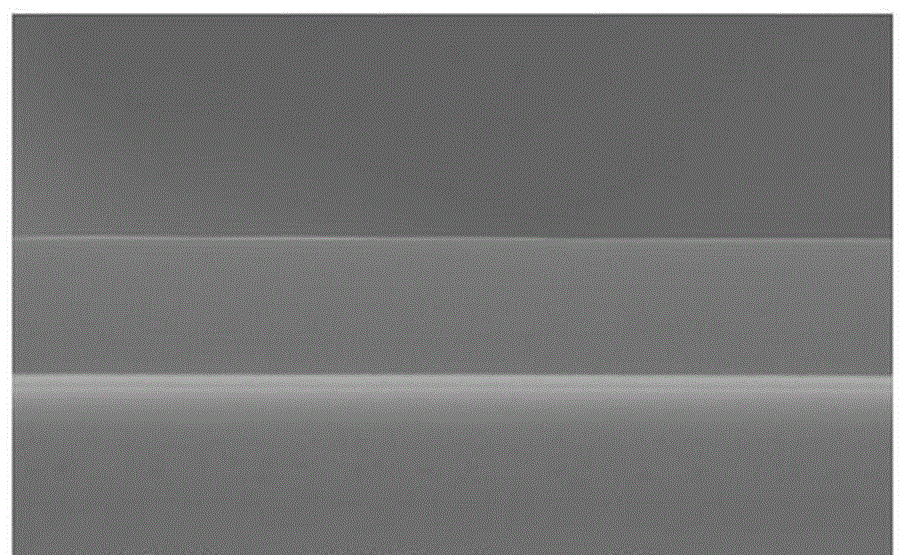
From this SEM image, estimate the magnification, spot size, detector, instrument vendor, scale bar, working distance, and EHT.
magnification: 9.495 K X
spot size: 3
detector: SE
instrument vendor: FEI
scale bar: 2000 nm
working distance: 10.6 mm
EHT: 15 kV